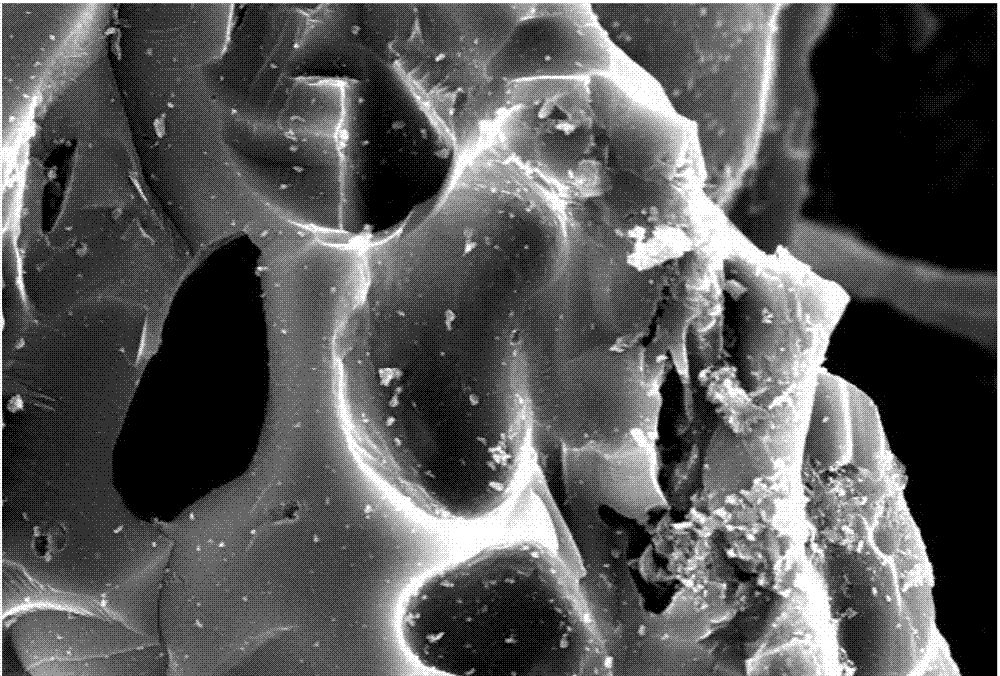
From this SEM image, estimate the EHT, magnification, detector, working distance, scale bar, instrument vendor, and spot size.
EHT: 20 kV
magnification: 1 K X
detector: SEI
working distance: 21 mm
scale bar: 10000 nm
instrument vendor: JEOL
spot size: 50